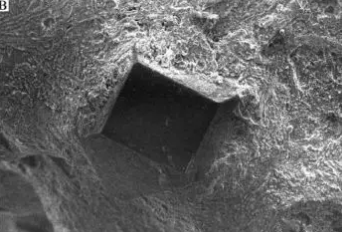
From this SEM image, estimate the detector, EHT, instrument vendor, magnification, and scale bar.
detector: SED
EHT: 20 kV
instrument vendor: other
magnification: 0.15 K X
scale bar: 300000 nm